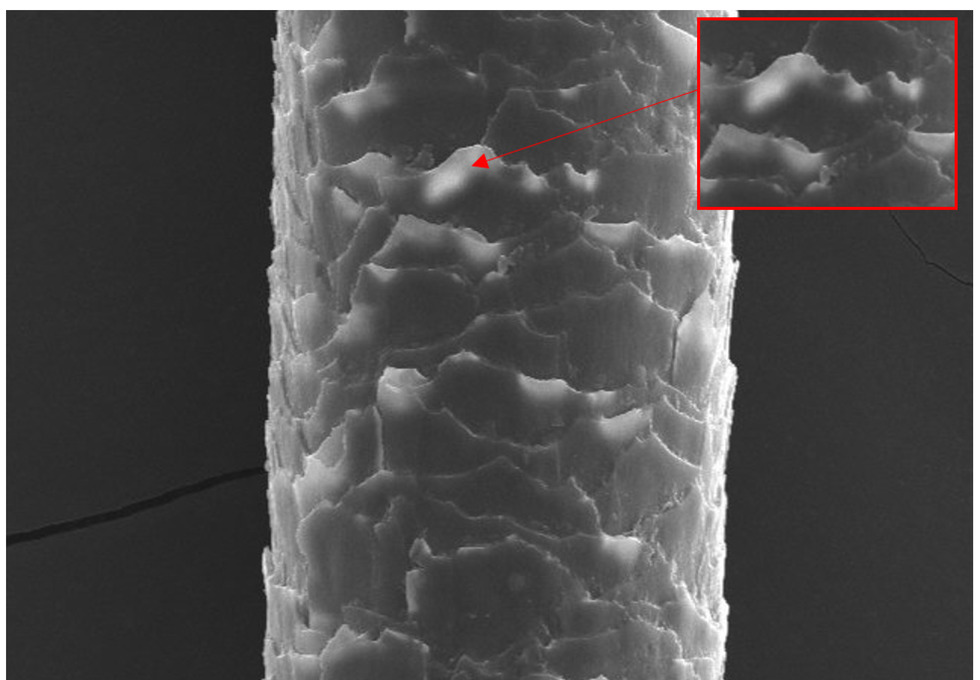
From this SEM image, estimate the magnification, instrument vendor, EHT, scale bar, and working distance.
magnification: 0.8 K X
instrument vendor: Hitachi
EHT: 15 kV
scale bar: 50000 nm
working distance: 13.1 mm